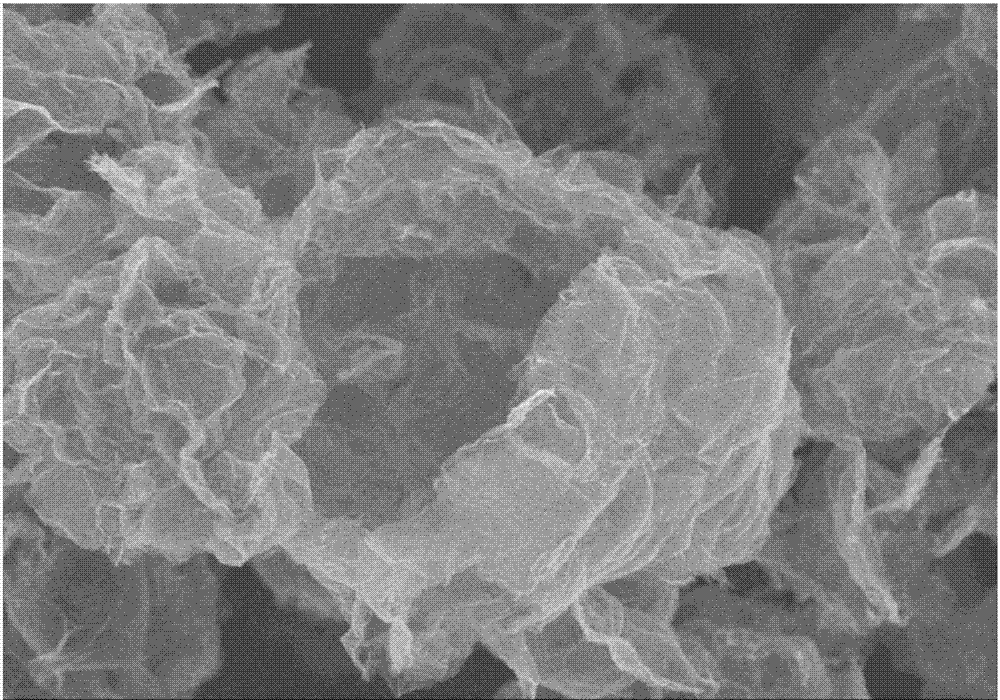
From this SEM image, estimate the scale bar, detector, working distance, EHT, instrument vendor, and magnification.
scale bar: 5000 nm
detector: SE(M)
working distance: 11 mm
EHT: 3 kV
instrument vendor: Hitachi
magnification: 8 K X